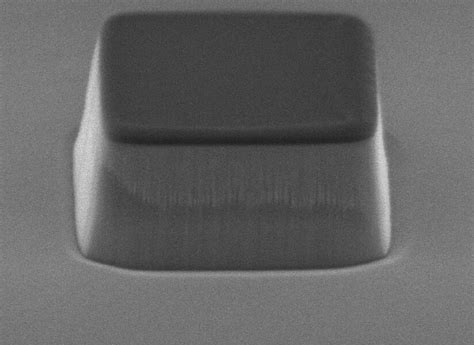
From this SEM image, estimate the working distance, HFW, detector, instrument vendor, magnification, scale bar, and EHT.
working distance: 9.7 mm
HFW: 3.66 µm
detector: SED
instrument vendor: JEOL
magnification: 35 K X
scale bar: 100 nm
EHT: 3 kV